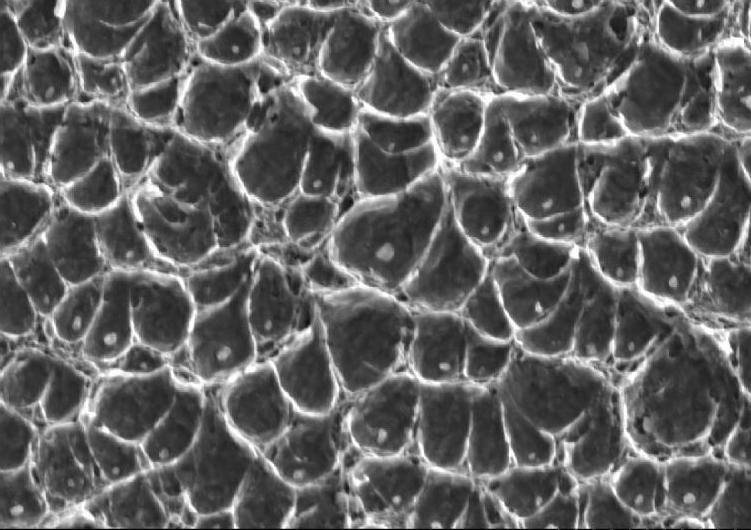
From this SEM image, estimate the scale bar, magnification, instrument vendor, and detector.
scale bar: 10000 nm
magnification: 3 K X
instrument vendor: Hitachi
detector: SE